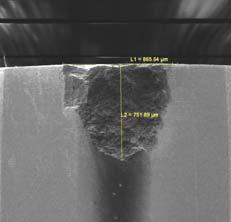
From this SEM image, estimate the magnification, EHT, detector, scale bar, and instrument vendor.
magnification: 0.119 K X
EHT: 5 kV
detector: SE Detector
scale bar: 500000 nm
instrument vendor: Tescan